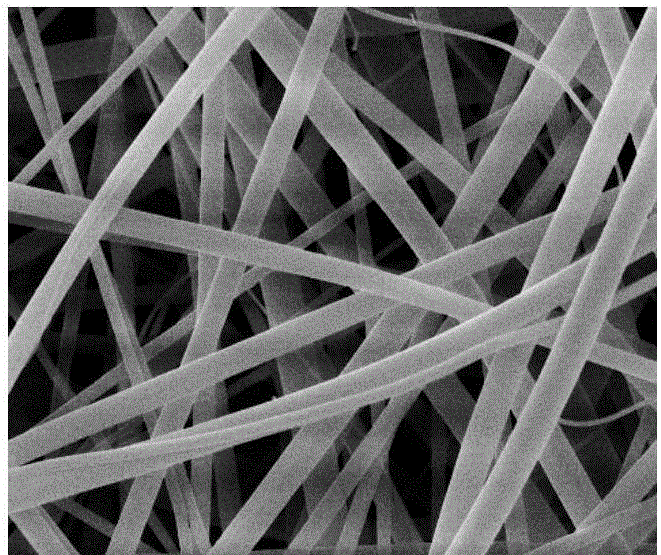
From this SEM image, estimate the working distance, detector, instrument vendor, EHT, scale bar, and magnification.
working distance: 10.6 mm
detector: ETD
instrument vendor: FEI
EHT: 10 kV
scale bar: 5000 nm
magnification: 20 K X